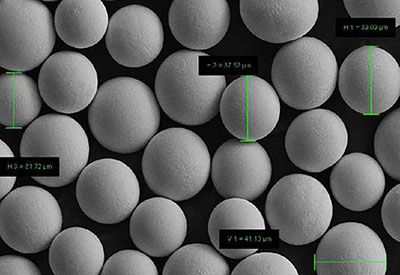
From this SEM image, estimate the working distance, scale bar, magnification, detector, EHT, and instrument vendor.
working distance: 7.6 mm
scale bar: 10000 nm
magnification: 0.5 K X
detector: SE2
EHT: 15 kV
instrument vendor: Zeiss